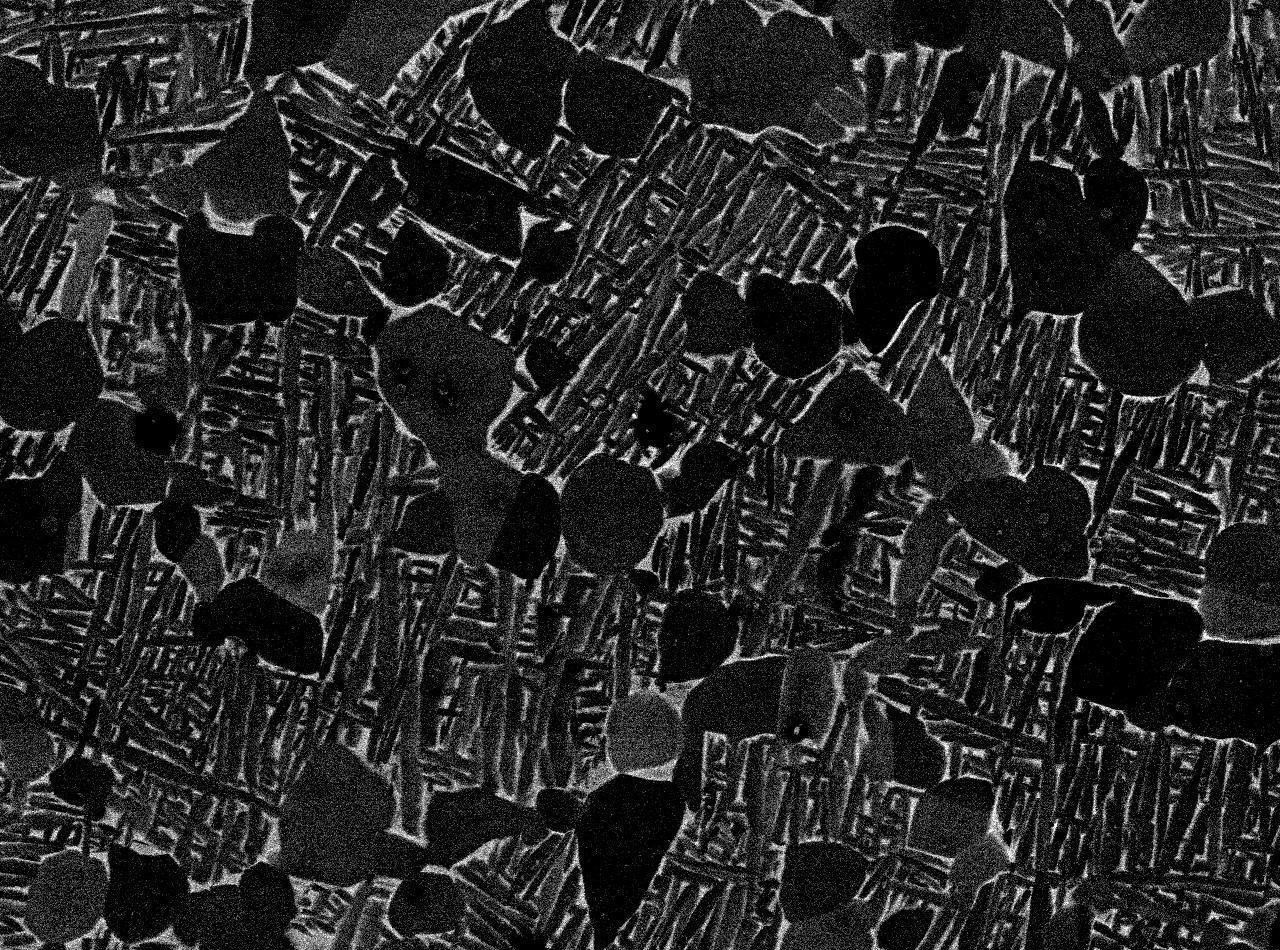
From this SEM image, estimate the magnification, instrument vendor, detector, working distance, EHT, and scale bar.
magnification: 1.5 K X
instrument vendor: JEOL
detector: COMPO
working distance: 10.1 mm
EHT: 15 kV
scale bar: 10000 nm